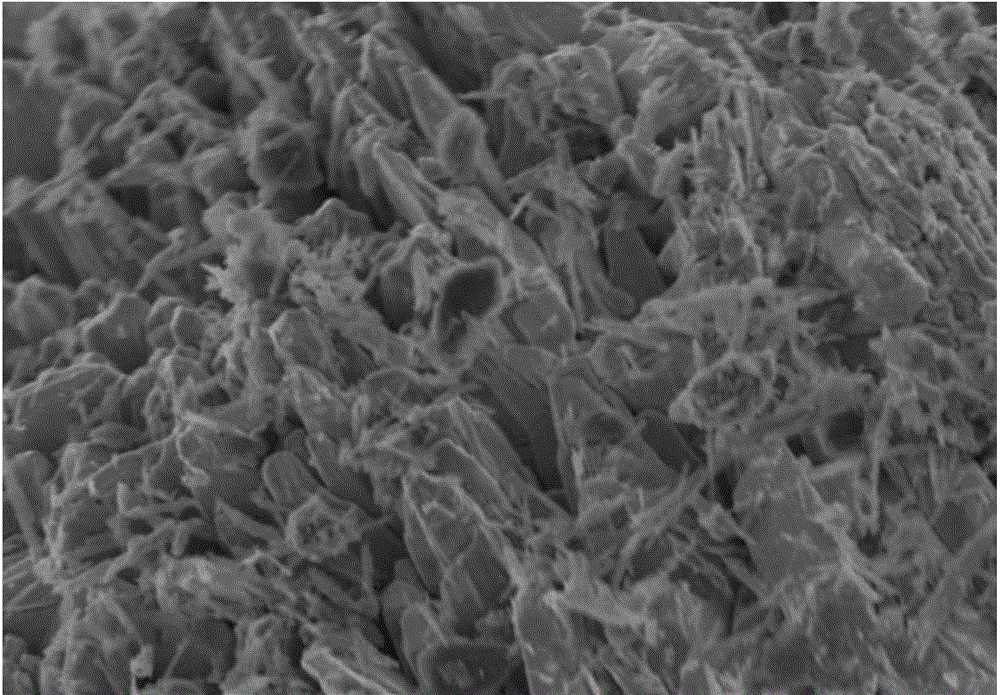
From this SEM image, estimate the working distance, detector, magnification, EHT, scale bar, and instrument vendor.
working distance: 5.6 mm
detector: SE(M)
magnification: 20 K X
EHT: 2 kV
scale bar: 2000 nm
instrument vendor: Hitachi